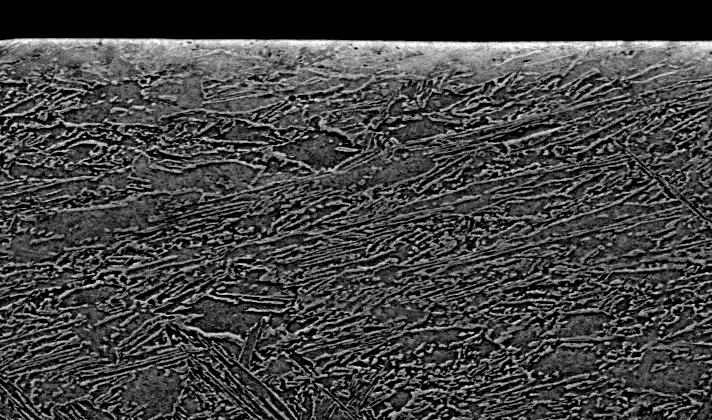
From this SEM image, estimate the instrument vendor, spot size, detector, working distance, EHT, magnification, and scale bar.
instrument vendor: FEI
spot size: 5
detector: BSE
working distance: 9.6 mm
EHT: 20 kV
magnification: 1.5 K X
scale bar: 20000 nm